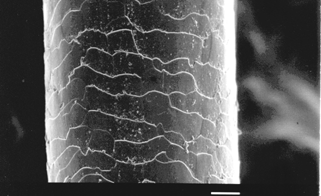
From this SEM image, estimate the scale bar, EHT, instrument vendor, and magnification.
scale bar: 10000 nm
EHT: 5 kV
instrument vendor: JEOL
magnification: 1 K X